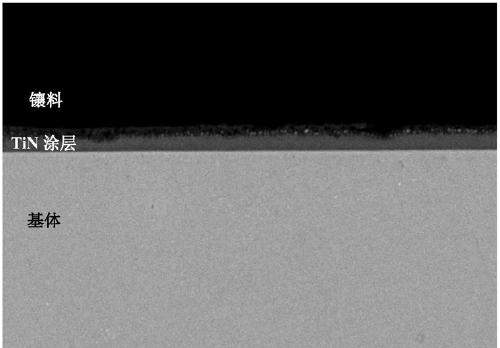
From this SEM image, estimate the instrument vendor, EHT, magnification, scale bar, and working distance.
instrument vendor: Hitachi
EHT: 20 kV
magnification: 2 K X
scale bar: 20000 nm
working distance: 11.8 mm